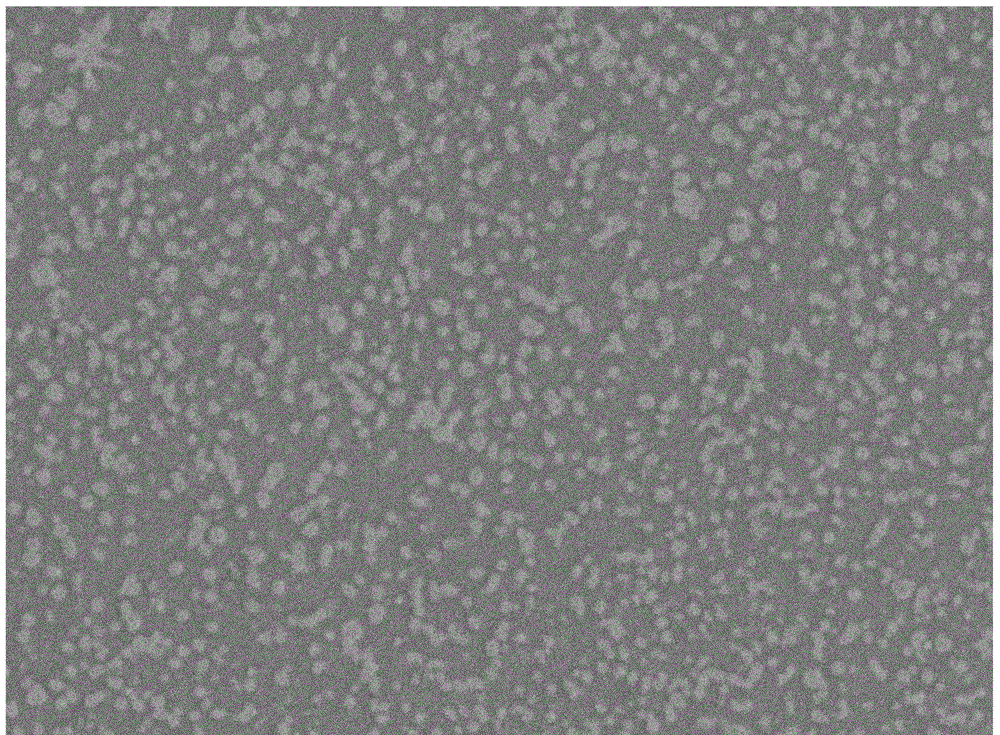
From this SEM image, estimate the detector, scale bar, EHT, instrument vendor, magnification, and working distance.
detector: SEI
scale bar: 10000 nm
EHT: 15 kV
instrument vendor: JEOL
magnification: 1.5 K X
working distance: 22 mm